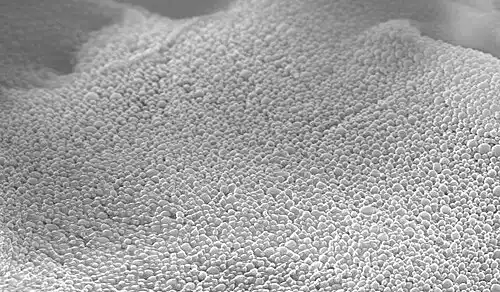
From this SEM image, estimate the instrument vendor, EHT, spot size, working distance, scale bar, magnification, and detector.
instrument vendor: FEI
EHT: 10 kV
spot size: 3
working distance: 8 mm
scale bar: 2000 nm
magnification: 11.405 K X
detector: SE